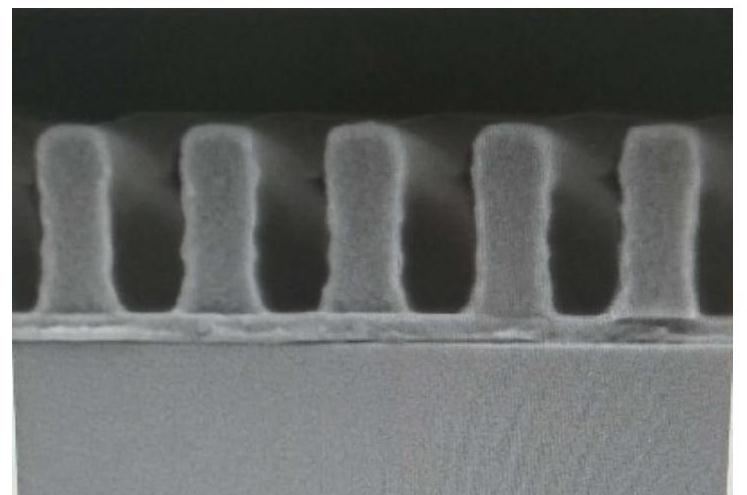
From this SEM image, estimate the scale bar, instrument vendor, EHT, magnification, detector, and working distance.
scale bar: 500 nm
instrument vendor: Hitachi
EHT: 1 kV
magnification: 100 K X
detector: SE(M)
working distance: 4 mm